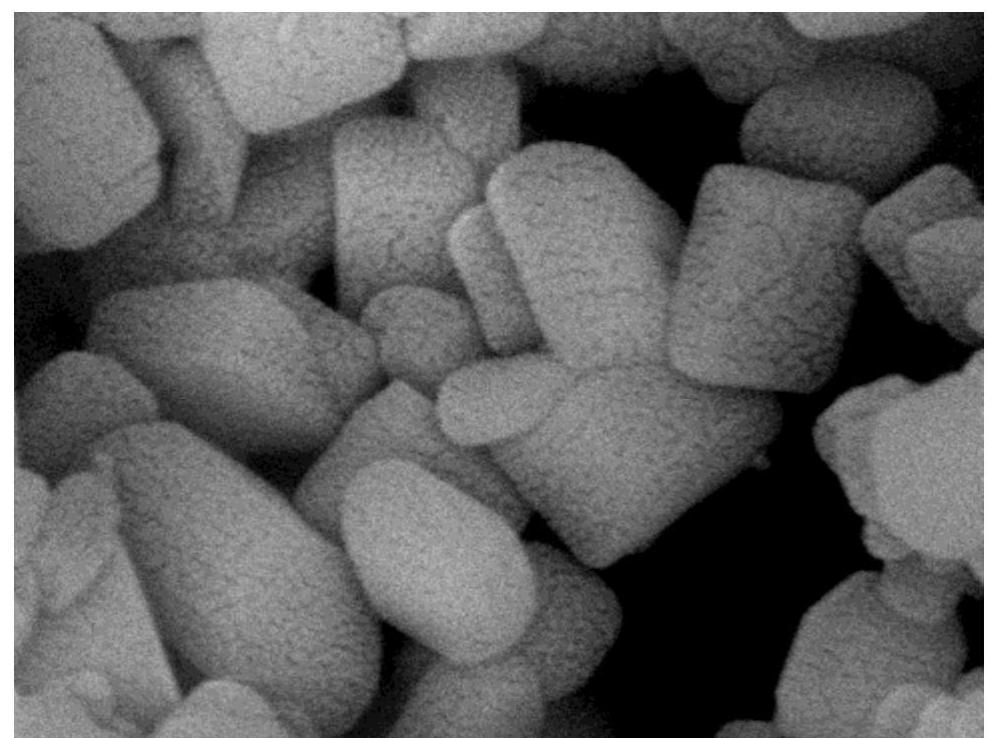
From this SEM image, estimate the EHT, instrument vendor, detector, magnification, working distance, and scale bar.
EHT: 8 kV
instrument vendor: JEOL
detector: SEI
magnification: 90 K X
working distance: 8.1 mm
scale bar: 100 nm